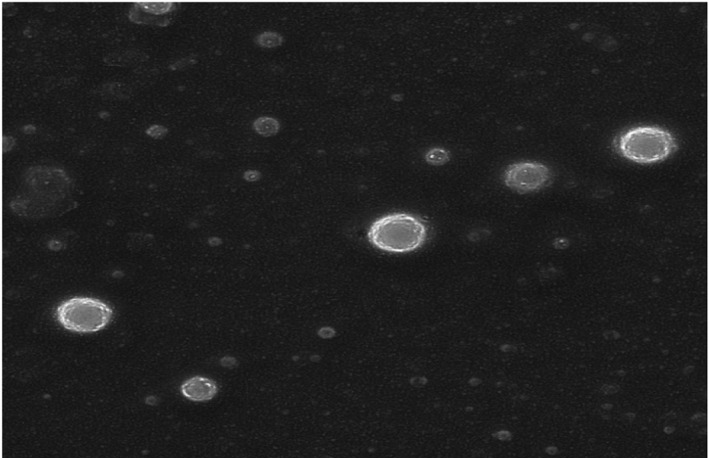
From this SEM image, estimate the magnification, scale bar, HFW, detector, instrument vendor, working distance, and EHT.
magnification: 8.58 K X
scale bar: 5000 nm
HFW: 16.1 µm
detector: InBeam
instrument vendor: Tescan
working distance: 4.85 mm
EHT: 10 kV